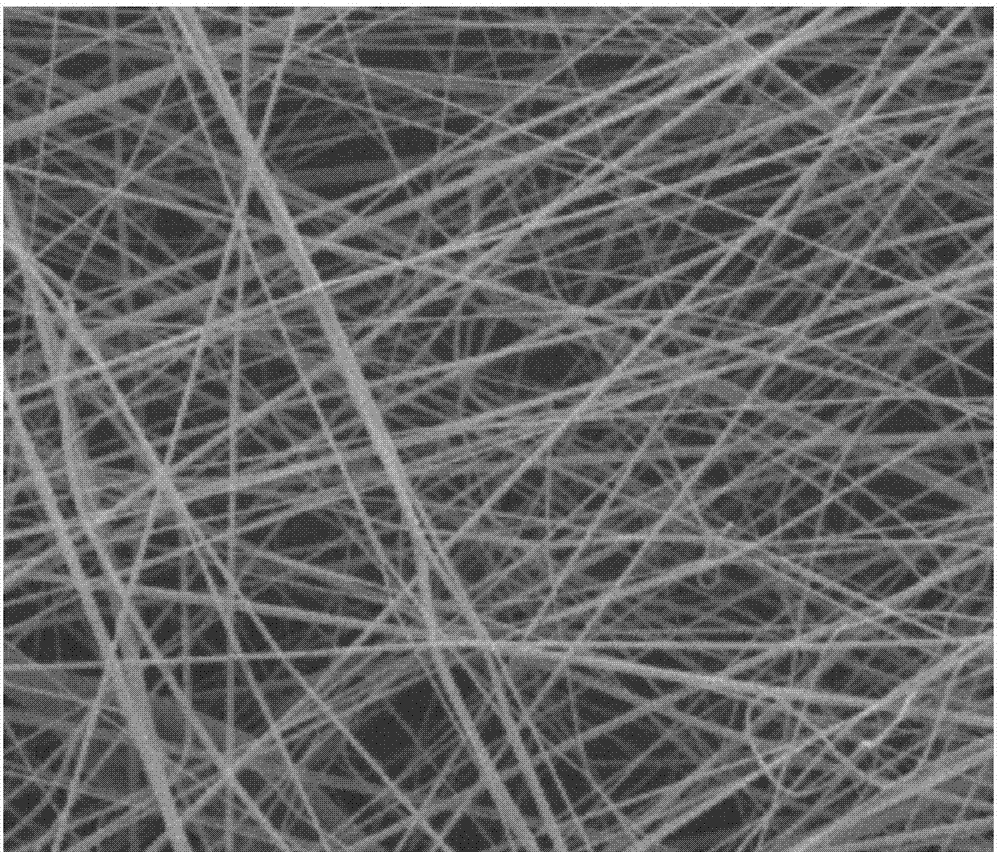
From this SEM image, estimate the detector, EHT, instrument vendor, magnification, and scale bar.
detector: ETD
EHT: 10 kV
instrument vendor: FEI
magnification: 5 K X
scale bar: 10000 nm